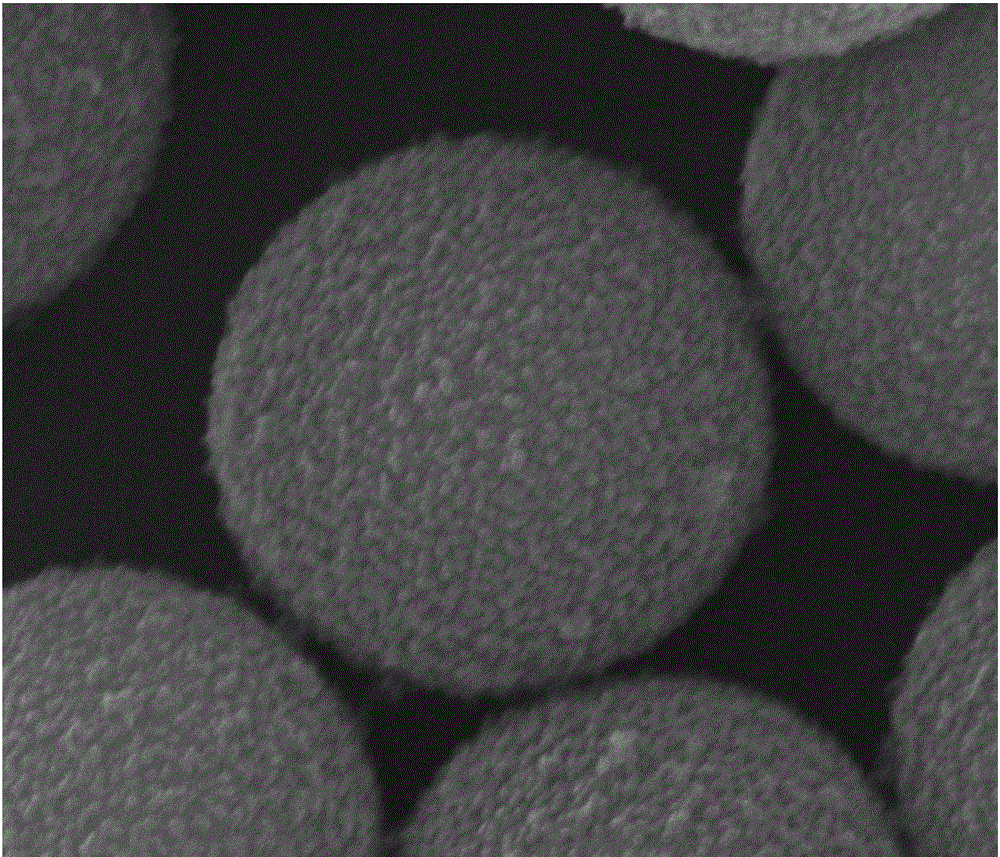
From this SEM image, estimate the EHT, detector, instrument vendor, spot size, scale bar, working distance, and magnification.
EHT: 25 kV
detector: ETD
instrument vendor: FEI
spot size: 3.5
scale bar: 4000 nm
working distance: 3.9 mm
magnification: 30 K X